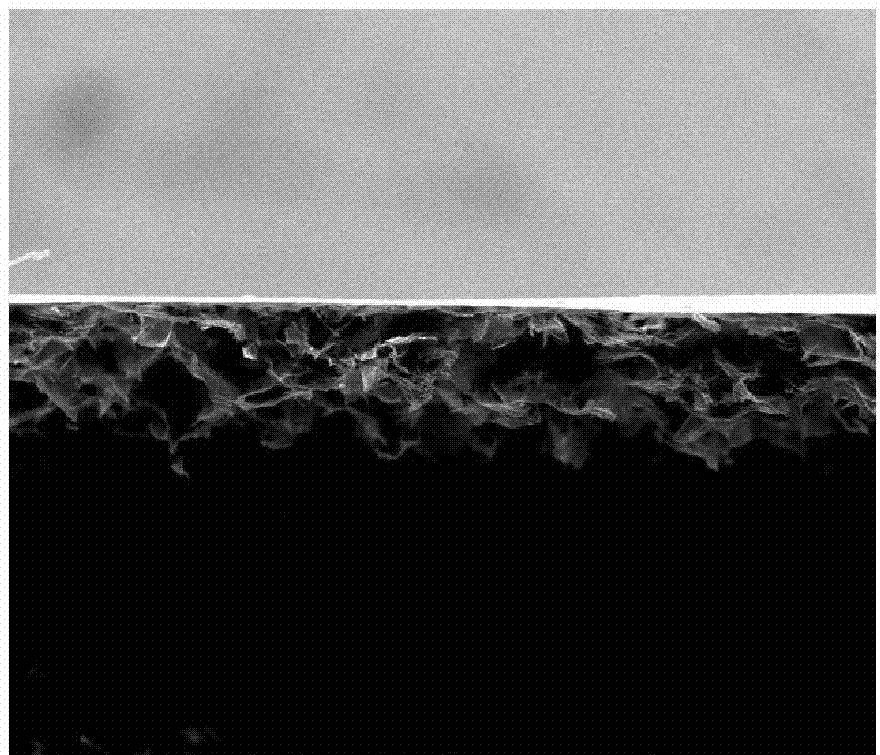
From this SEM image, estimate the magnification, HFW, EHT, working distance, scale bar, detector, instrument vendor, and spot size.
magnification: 0.4 K X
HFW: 746 µm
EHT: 20 kV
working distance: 11.4 mm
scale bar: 300000 nm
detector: ETD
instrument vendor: FEI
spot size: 3